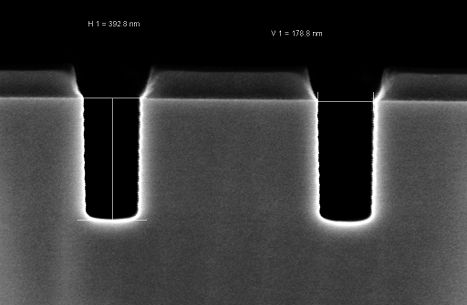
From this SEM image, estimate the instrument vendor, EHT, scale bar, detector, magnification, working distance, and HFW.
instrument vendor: Zeiss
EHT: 2 kV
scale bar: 100 nm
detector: InLens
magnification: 200 K X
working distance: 3.2 mm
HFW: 1.501 µm